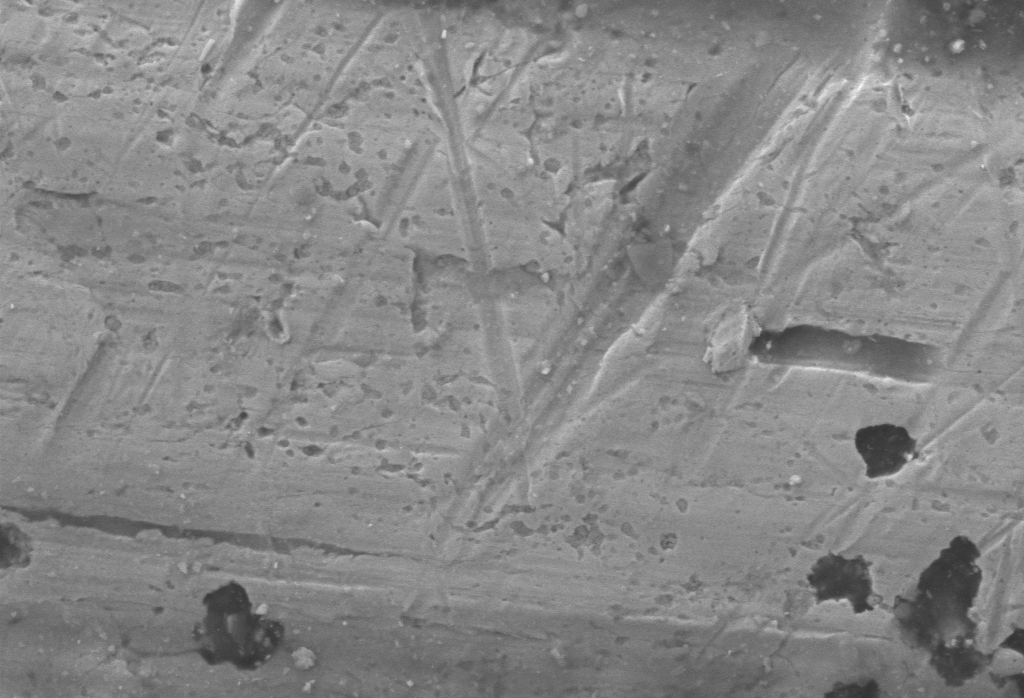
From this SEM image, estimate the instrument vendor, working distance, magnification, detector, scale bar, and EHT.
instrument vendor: Zeiss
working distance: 7.1 mm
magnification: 20 K X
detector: SESI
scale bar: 1000 nm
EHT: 5 kV